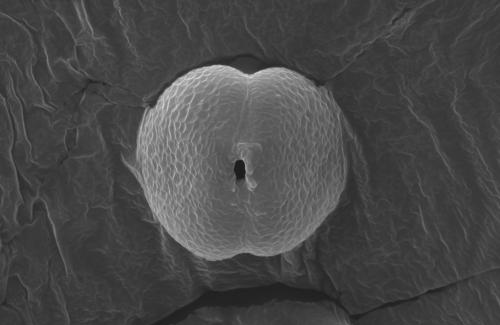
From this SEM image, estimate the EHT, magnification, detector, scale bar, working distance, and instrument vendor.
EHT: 12.5 kV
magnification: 15 K X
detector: ETD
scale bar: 5000 nm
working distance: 10.2 mm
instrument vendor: FEI